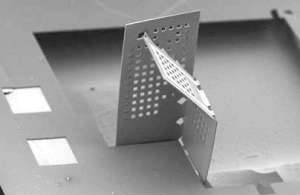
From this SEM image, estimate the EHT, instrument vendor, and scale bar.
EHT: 10 kV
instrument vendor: JEOL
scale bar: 500000 nm